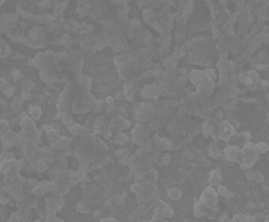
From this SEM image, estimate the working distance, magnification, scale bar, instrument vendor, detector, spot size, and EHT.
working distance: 11.1 mm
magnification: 1 K X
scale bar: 50000 nm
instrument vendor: FEI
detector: ETD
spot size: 5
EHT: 30 kV